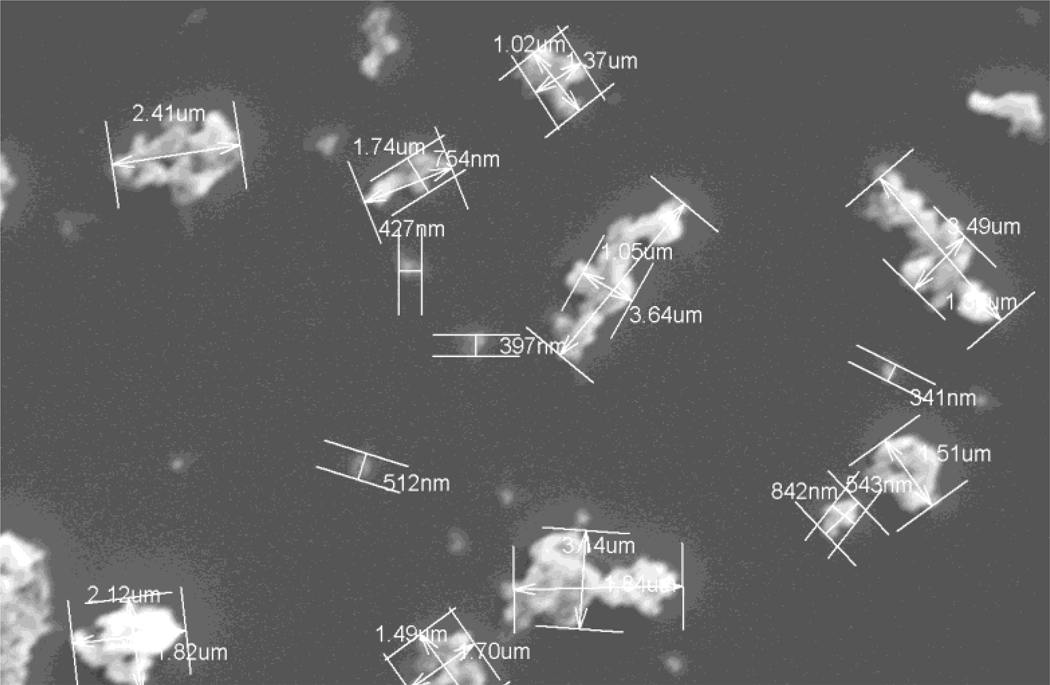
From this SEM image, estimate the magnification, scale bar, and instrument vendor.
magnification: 6.5 K X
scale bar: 5000 nm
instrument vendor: Hitachi